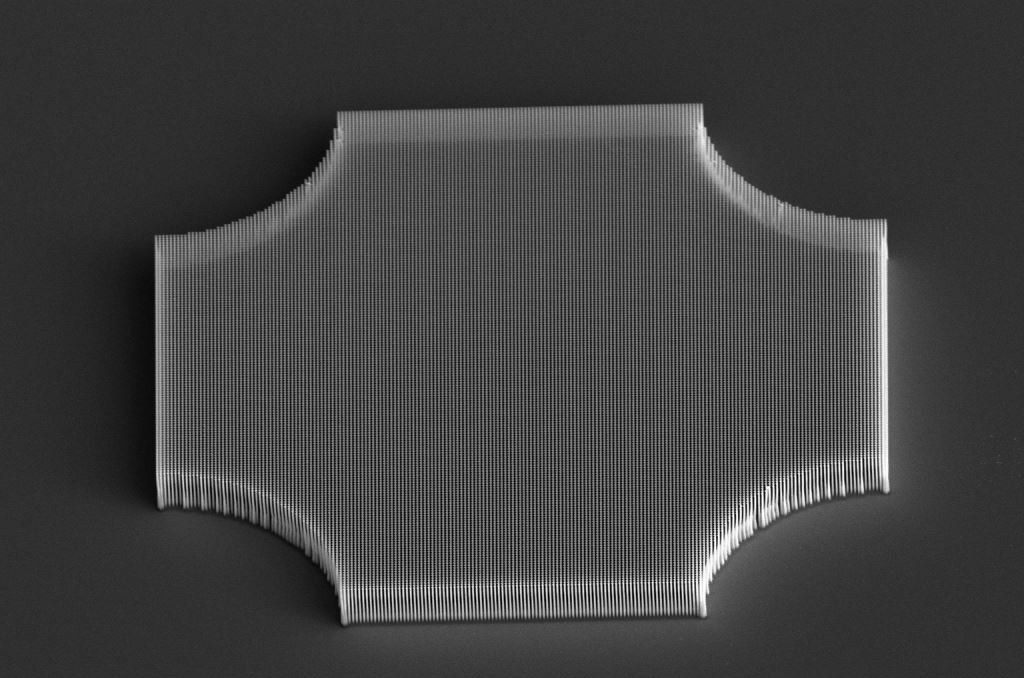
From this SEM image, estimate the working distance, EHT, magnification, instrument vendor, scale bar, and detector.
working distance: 22.8 mm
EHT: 10 kV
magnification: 1.5 K X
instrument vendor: FEI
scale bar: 40000 nm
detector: ETD